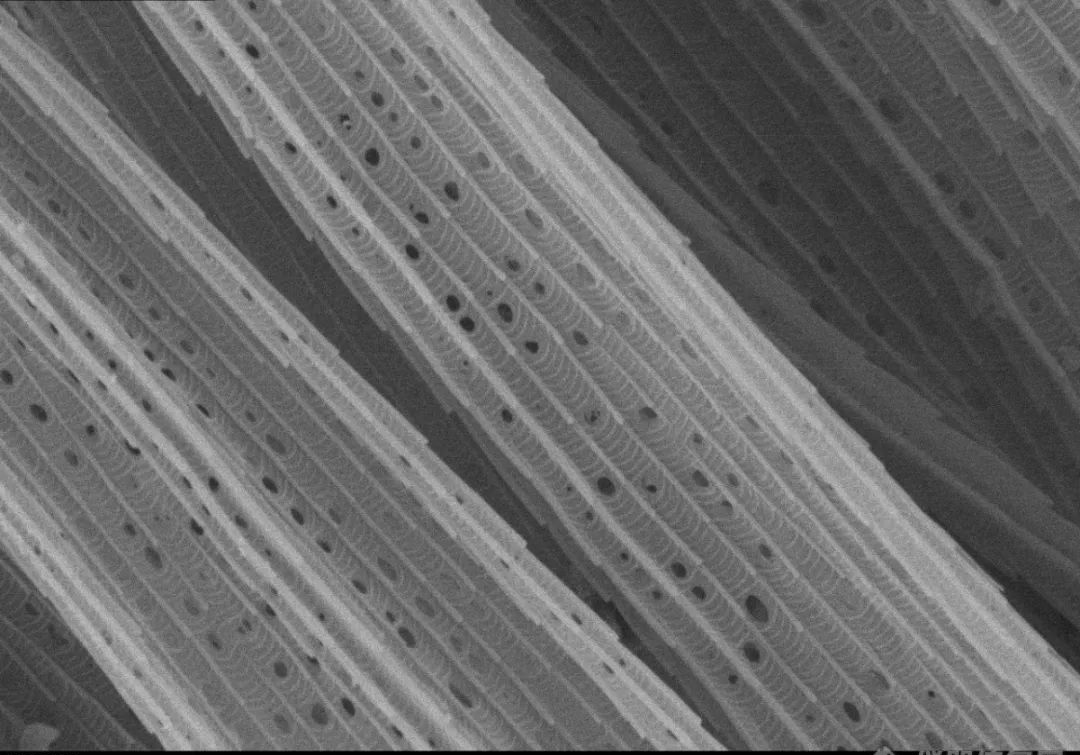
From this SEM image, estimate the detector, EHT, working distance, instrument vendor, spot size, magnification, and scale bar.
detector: SEI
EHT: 7 kV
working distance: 5.1 mm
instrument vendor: other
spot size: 13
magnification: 10 K X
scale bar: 1000 nm